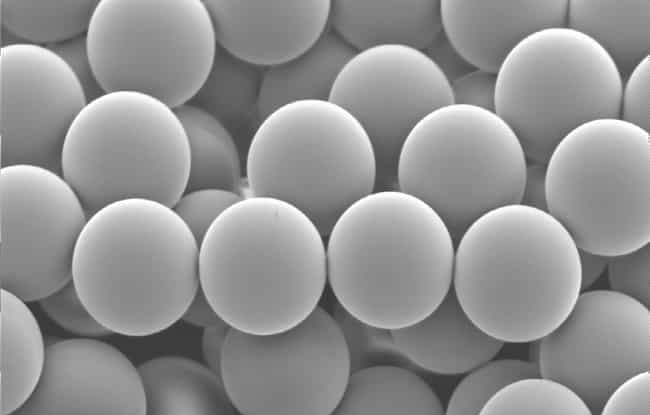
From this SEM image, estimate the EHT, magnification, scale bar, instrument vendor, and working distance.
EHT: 5 kV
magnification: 5 K X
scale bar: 1000 nm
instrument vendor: JEOL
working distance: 18 mm